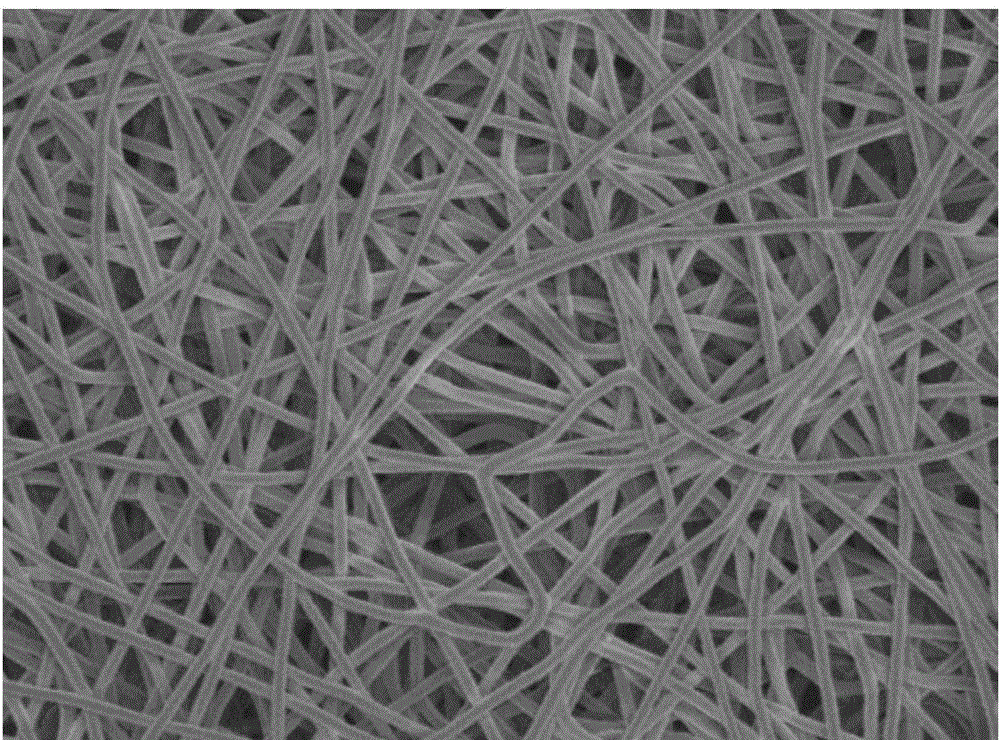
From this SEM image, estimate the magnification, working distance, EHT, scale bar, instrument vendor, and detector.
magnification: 1 K X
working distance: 10.6 mm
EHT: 5 kV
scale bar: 10000 nm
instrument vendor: JEOL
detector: SEI